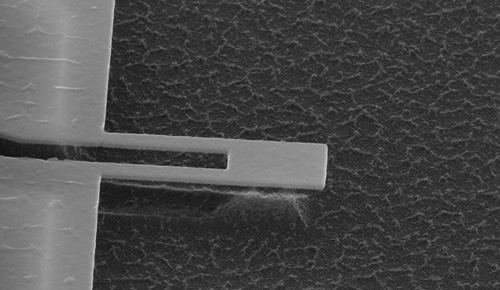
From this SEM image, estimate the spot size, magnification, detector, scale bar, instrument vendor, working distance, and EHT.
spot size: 3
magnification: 20 K X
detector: TLD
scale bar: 1000 nm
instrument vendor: FEI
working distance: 5 mm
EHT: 20 kV